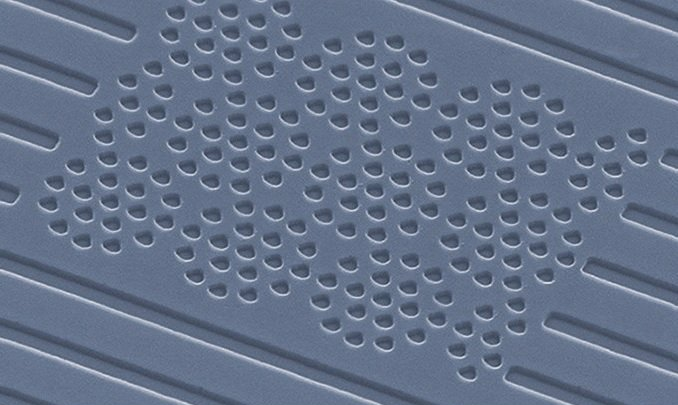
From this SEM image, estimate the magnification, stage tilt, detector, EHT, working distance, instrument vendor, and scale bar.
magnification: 14.19 K X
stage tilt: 44.6°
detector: SE2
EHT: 20 kV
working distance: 8 mm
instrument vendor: Zeiss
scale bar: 1000 nm